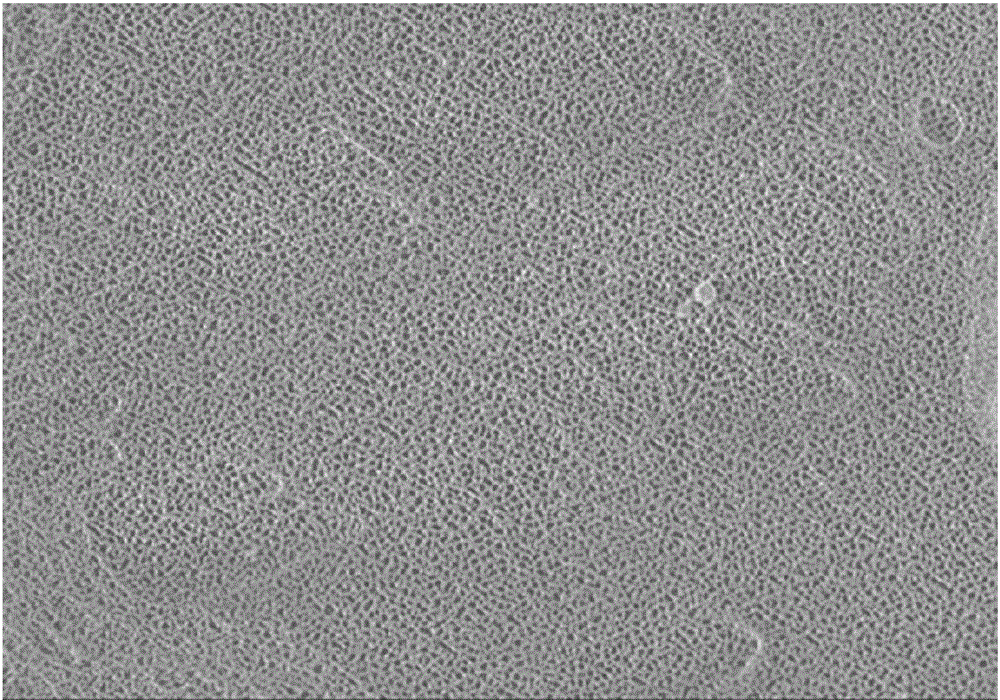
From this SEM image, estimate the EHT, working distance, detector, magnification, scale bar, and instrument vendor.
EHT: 1.5 kV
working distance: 3.8 mm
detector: SE(M,LA0)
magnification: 20 K X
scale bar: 2000 nm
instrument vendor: Hitachi